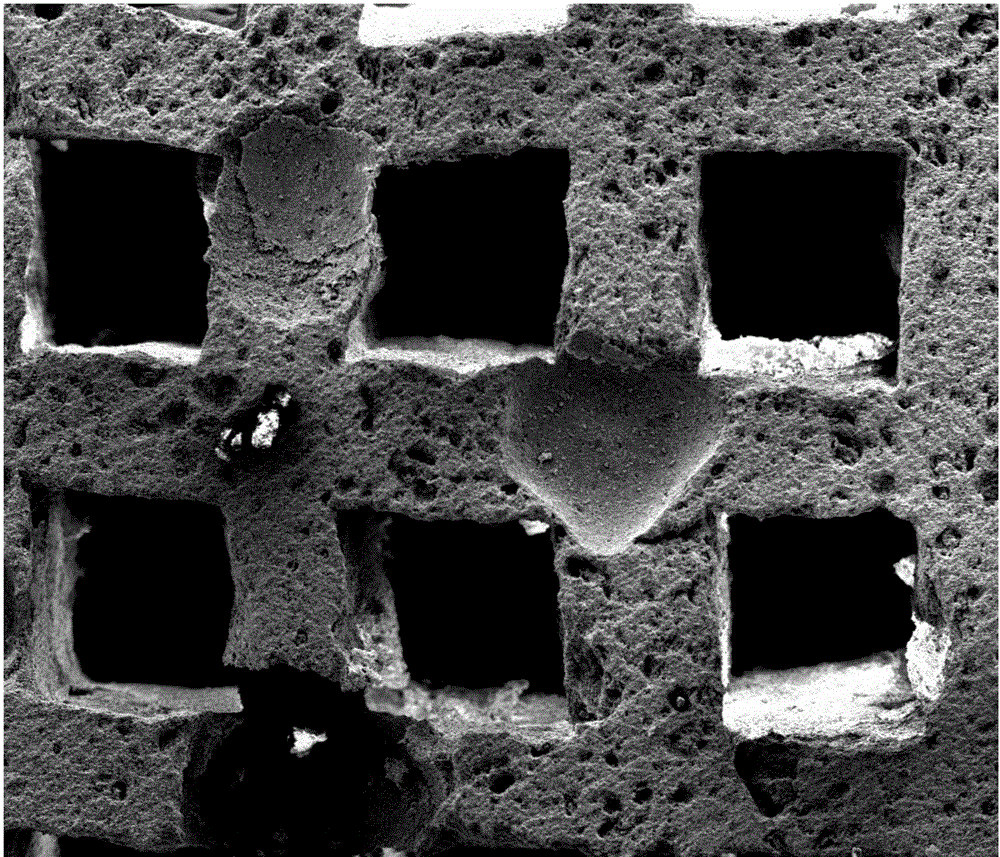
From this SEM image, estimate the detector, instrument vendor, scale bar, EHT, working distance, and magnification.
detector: ETD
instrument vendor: FEI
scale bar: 500000 nm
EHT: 12.5 kV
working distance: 15.2 mm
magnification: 0.15 K X